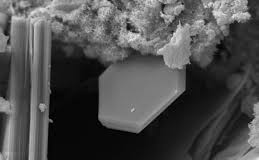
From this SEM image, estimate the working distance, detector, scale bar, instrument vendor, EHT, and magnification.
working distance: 6.5 mm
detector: SE1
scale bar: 3000 nm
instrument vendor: Zeiss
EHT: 18 kV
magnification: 6 K X